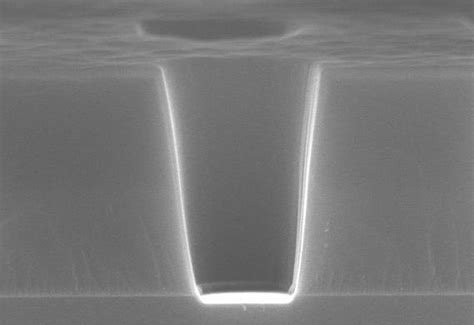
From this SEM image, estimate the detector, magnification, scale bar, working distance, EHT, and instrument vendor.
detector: SE(U)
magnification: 60 K X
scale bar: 500 nm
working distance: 8.3 mm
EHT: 15 kV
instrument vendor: Hitachi